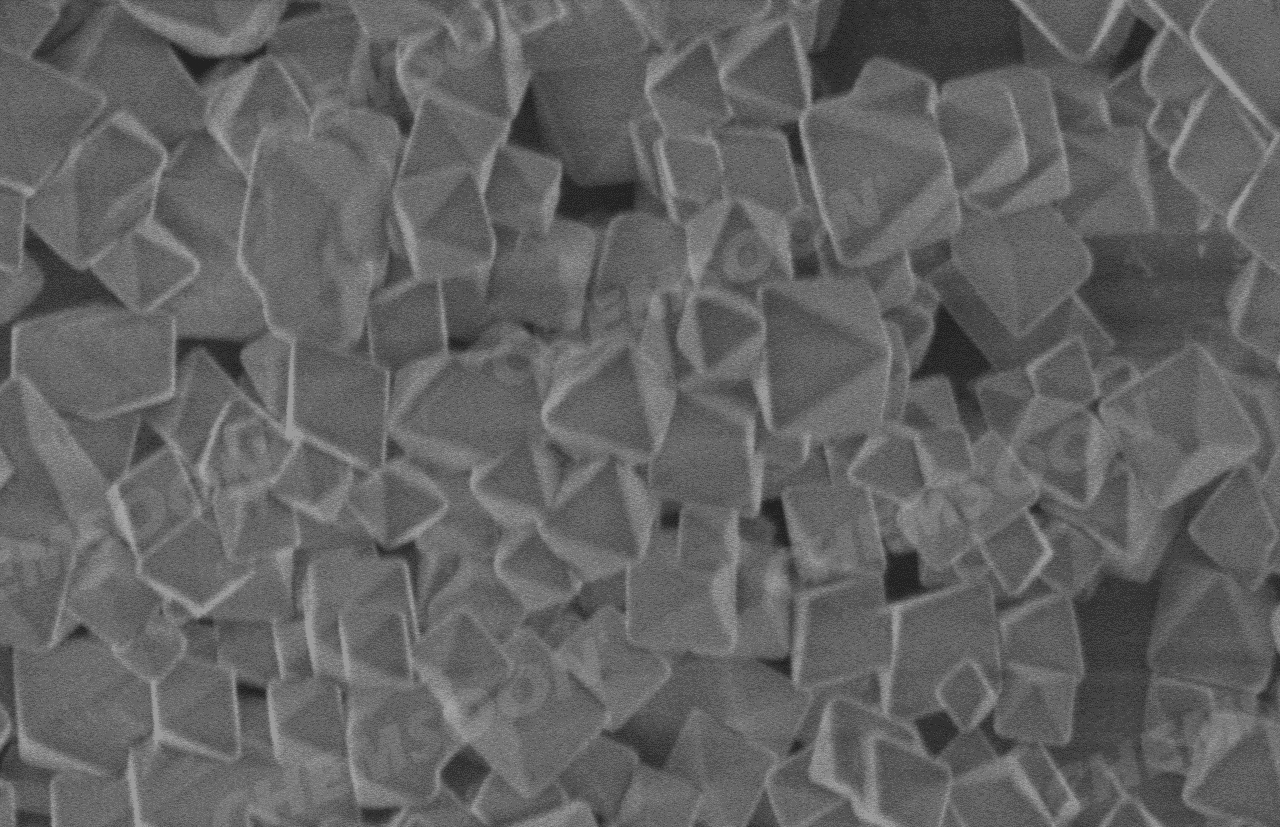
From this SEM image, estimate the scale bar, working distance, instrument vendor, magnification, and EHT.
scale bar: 2000 nm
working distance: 4.8 mm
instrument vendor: Hitachi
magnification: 20 K X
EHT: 1 kV